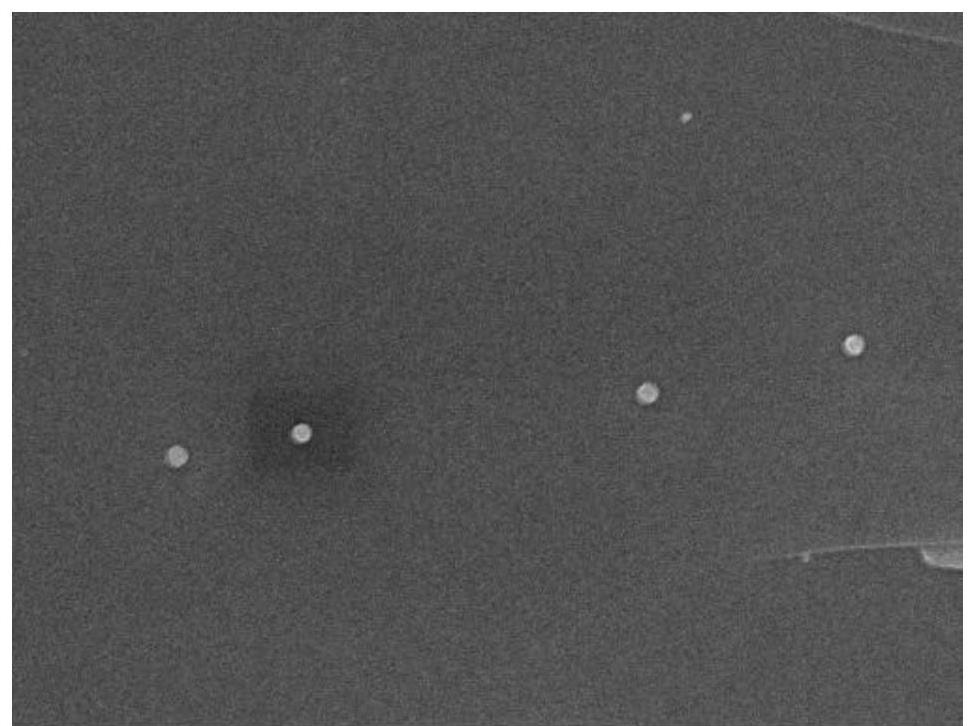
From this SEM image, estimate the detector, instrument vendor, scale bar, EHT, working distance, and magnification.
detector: SEI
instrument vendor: JEOL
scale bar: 1000 nm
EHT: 8 kV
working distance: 7.7 mm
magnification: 15 K X